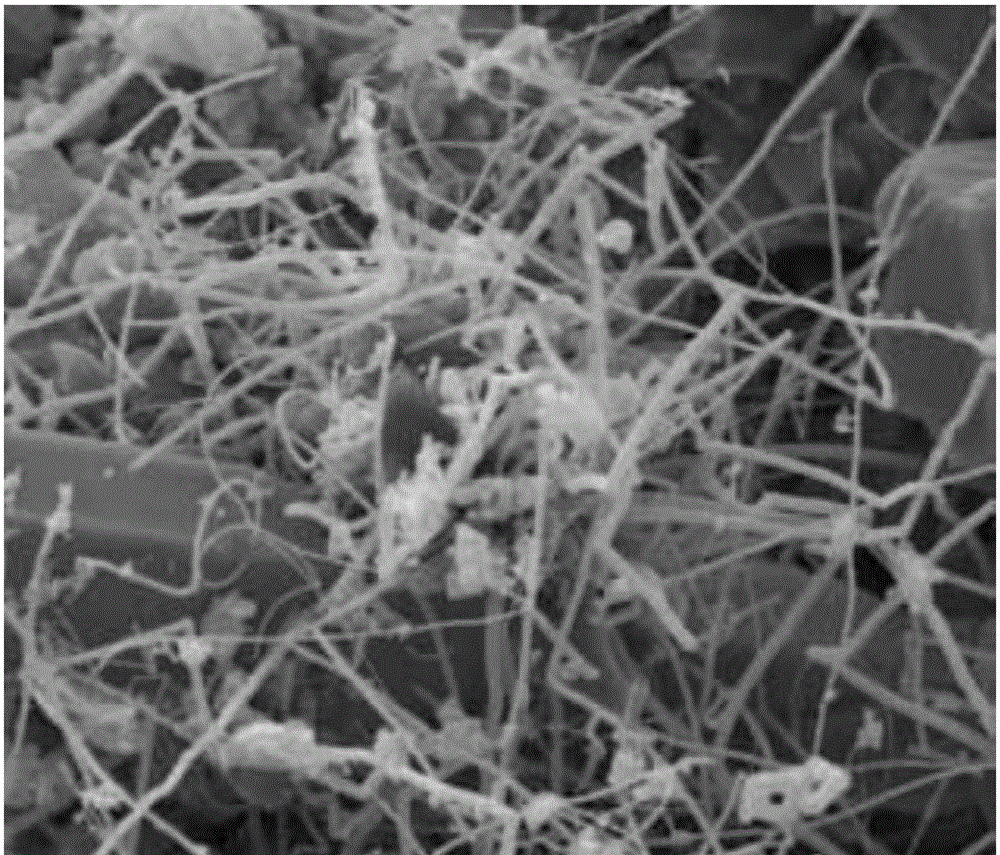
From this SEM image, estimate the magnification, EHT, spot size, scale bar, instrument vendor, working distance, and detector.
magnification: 3 K X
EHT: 15 kV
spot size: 4.5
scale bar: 10000 nm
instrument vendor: FEI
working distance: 11.4 mm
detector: ETD